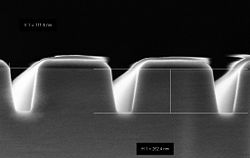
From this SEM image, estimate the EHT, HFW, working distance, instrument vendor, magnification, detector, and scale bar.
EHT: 2 kV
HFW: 1.566 µm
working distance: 2.2 mm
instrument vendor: Zeiss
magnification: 200 K X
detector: InLens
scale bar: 200 nm